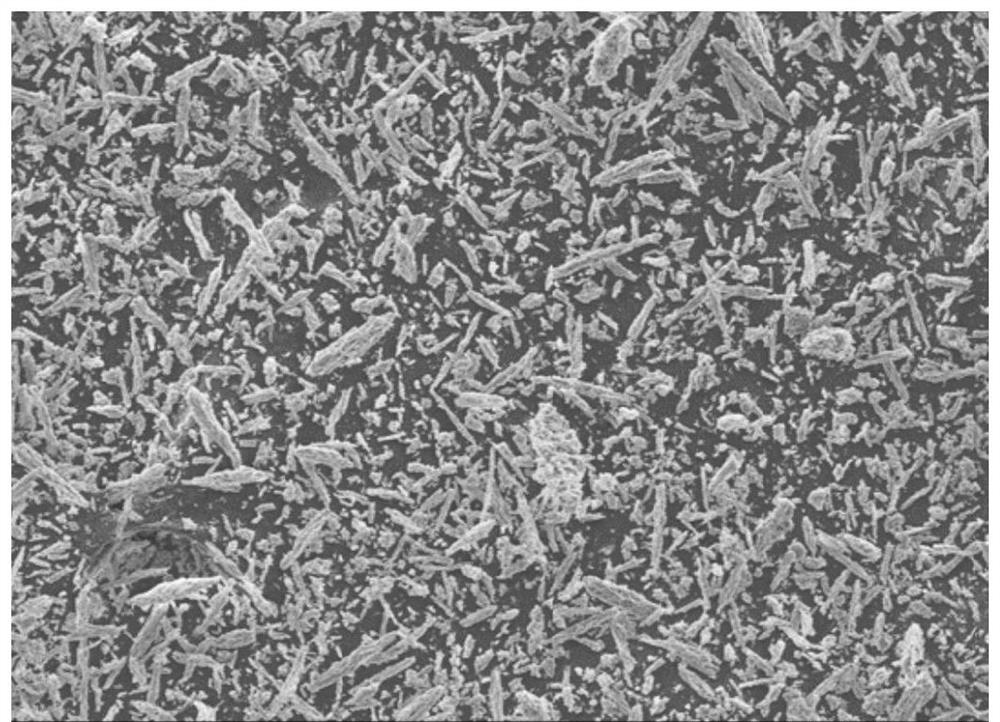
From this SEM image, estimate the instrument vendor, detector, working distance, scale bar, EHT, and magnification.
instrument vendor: Hitachi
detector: SE(M)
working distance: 8.7 mm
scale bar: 200000 nm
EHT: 5 kV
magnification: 0.2 K X